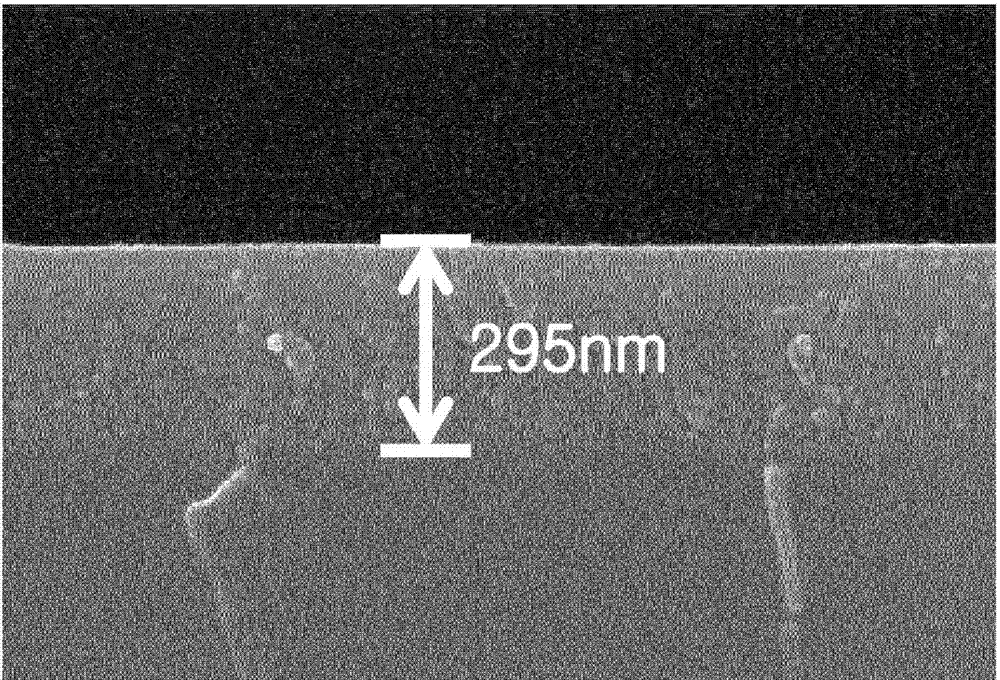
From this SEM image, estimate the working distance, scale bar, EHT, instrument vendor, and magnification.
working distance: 12.8 mm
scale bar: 500 nm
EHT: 15 kV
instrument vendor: Hitachi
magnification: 100 K X